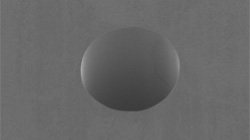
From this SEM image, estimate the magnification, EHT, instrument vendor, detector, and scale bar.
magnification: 0.45 K X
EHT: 15 kV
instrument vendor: JEOL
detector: SEI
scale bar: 50000 nm